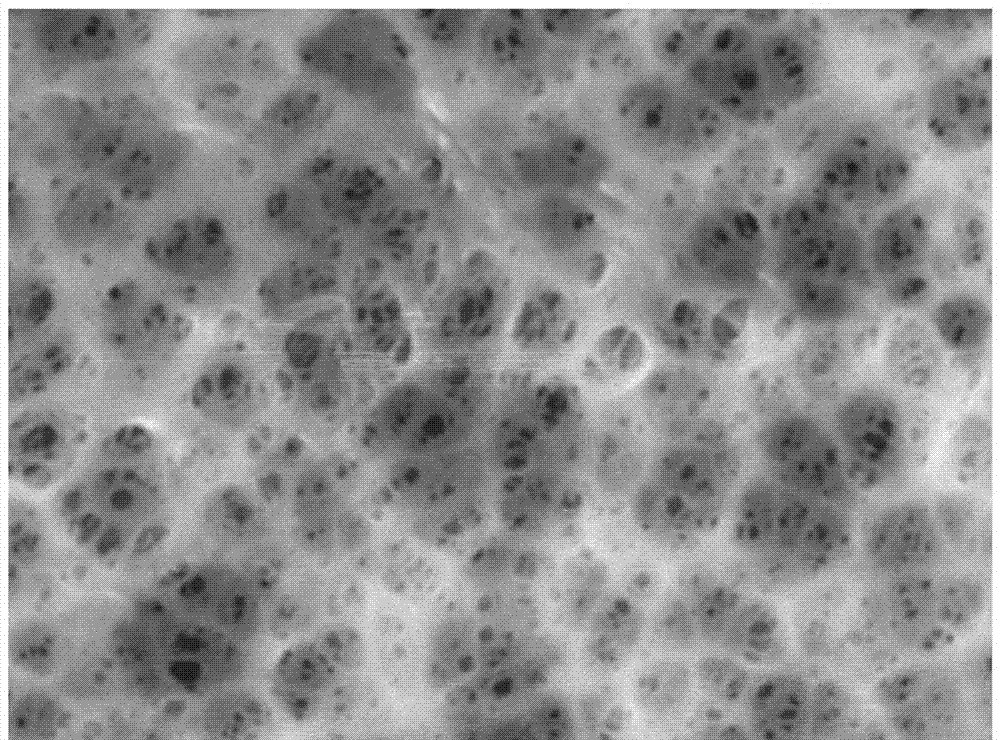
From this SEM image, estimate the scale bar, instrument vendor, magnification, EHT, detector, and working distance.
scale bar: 1000 nm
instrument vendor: JEOL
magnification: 5 K X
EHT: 20 kV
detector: SEI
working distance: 9.4 mm